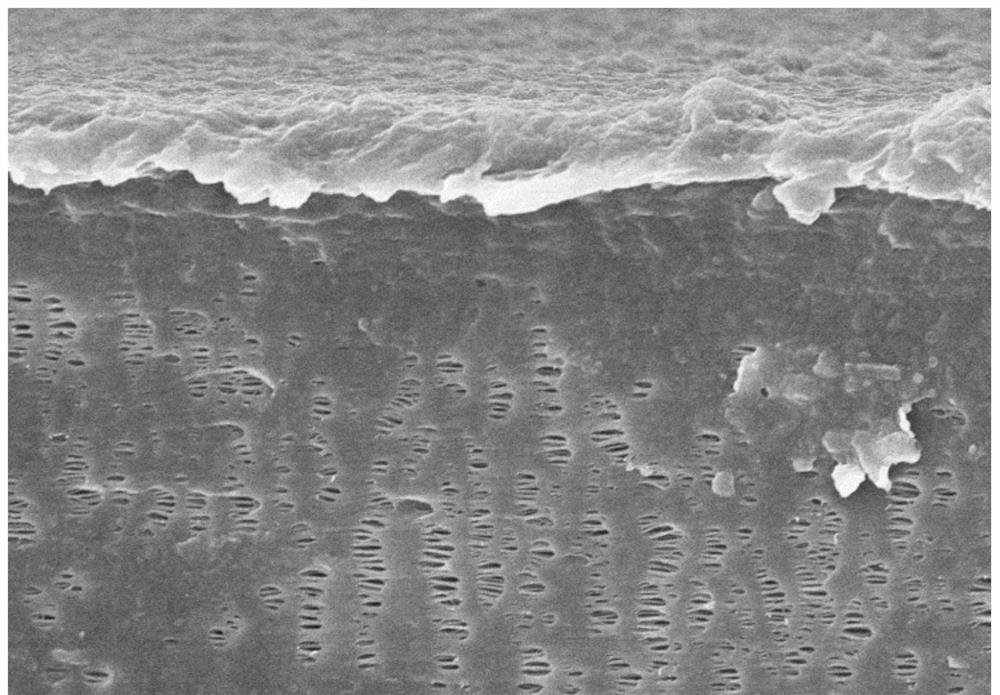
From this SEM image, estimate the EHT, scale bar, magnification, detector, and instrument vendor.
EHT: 8 kV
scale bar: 5000 nm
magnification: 7 K X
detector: SE(M)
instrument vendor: Hitachi